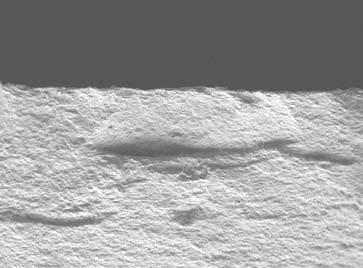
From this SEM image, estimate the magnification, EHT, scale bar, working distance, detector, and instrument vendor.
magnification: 0.3 K X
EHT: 5 kV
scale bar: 10000 nm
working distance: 7.2 mm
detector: LEI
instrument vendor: JEOL